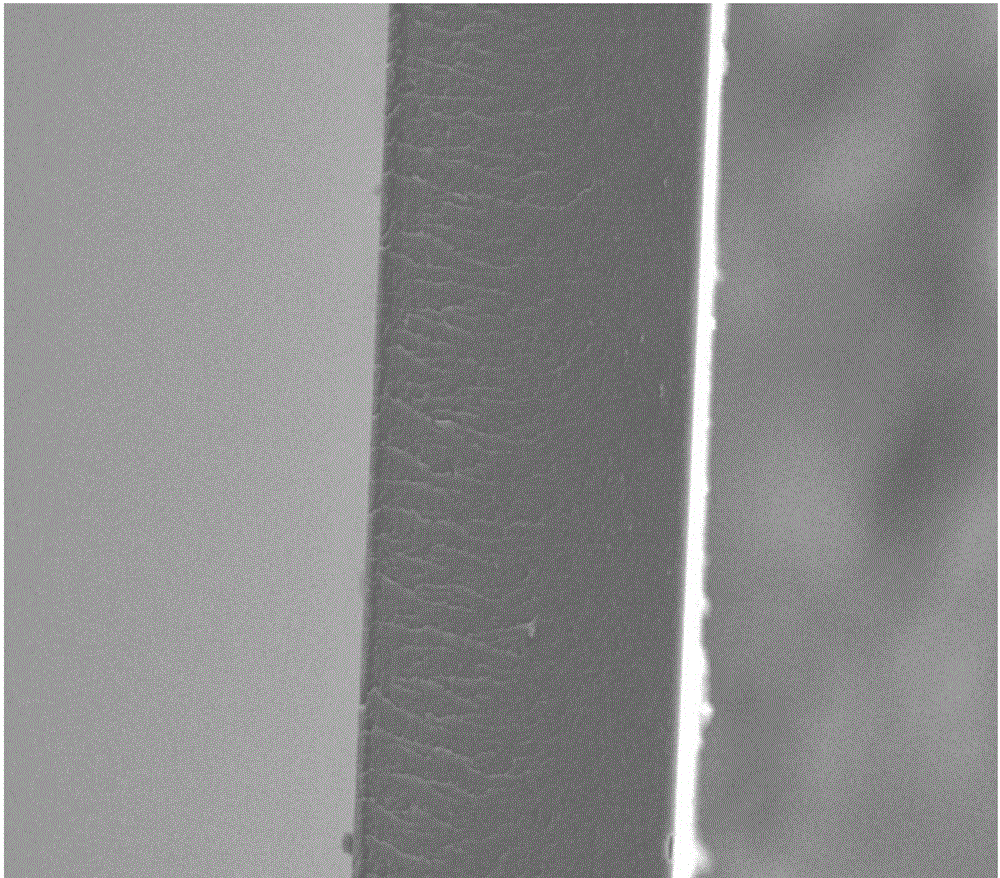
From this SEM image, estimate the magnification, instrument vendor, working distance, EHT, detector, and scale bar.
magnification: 2 K X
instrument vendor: FEI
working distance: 12.2 mm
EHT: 10 kV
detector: ETD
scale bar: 50000 nm